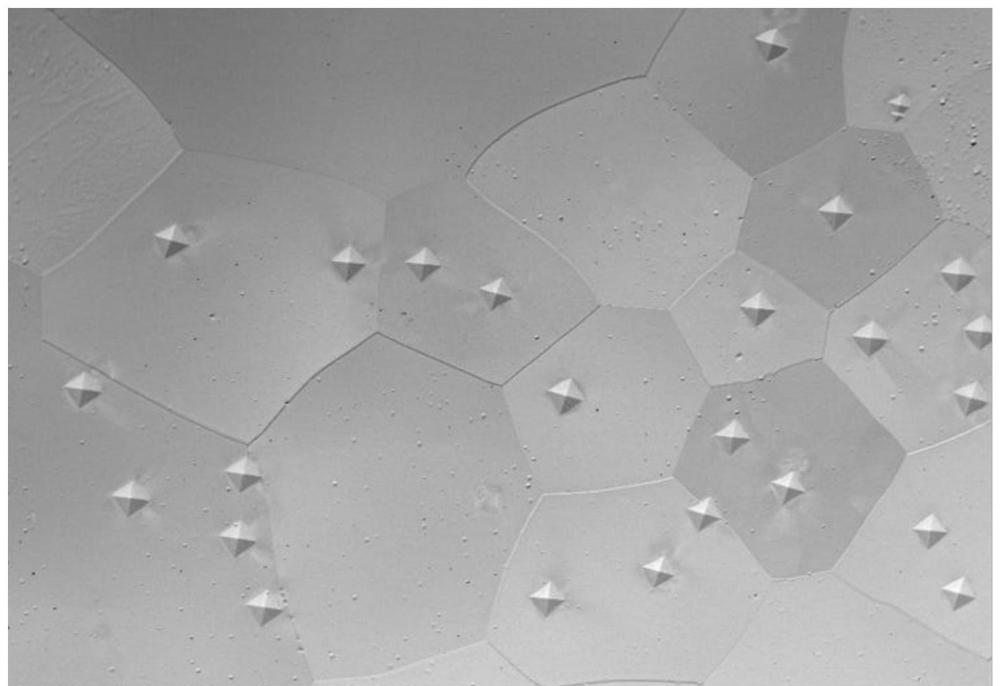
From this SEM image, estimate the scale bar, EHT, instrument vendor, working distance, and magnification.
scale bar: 500000 nm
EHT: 20 kV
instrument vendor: FEI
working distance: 10.9 mm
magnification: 0.15 K X